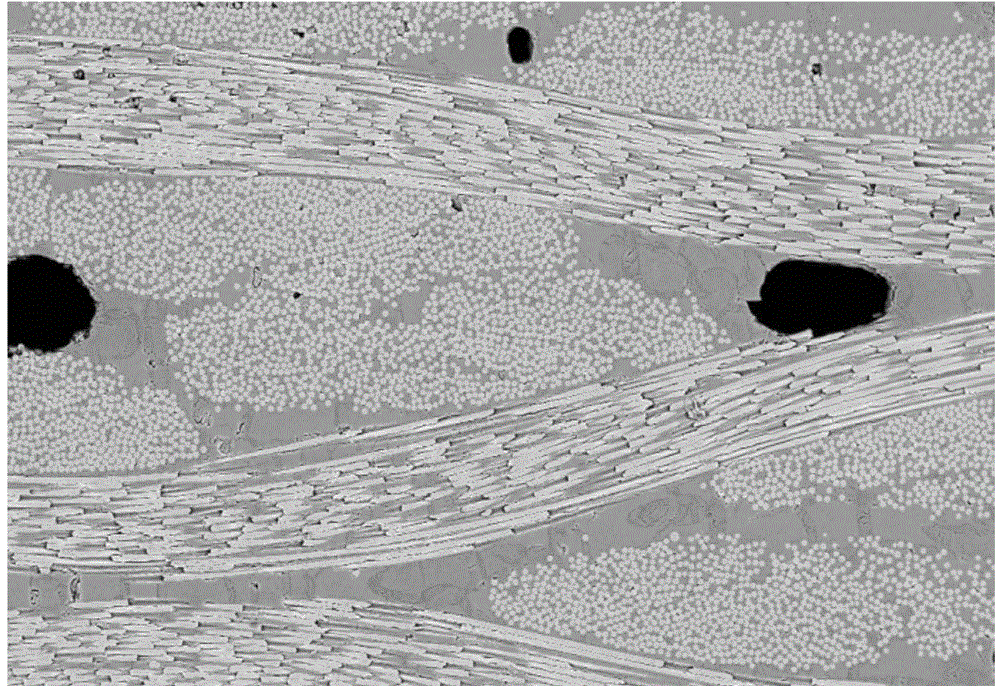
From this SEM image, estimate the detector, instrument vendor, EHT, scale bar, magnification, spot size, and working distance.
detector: DualBSD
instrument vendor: FEI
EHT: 20 kV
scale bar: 500000 nm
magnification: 0.2 K X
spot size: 6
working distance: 9.7 mm